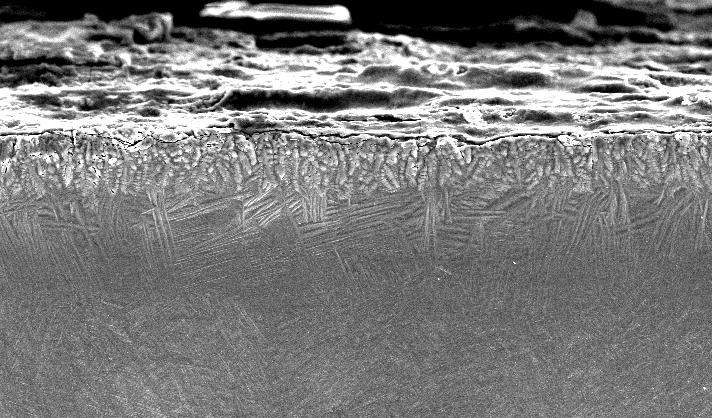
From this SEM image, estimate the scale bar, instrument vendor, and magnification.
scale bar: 100000 nm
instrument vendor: FEI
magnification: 0.25 K X